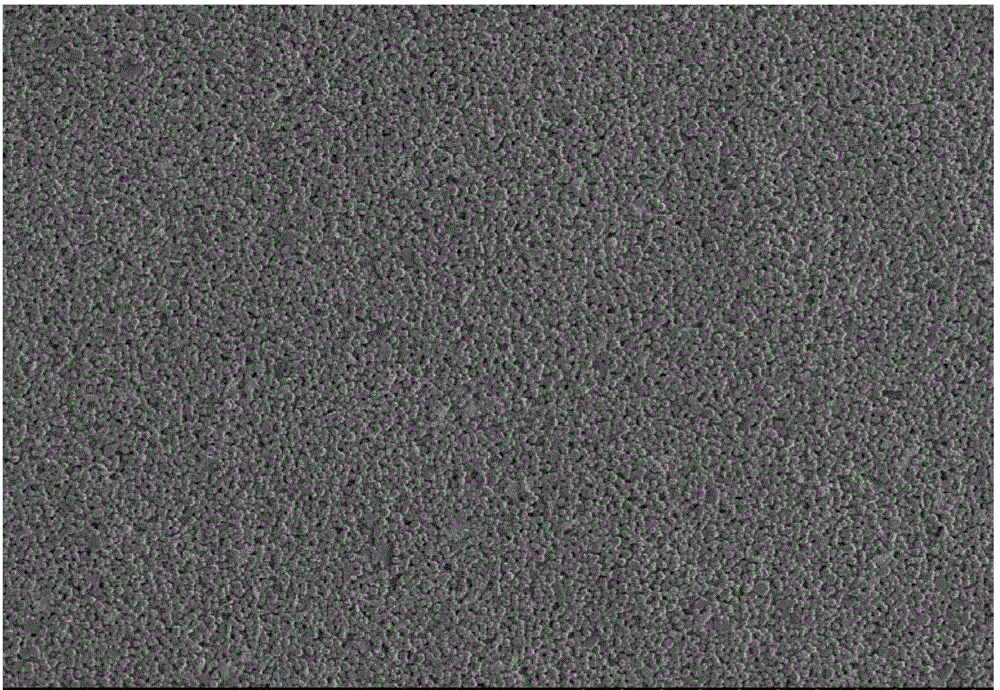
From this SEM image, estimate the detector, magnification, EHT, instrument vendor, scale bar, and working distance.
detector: SE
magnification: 0.1 K X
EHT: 15 kV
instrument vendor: Hitachi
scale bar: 500000 nm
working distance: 12.8 mm